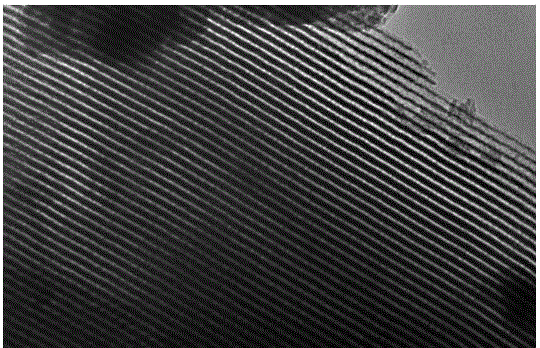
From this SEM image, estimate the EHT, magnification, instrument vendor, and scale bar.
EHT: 200 kV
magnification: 100 K X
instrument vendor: JEOL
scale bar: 100 nm